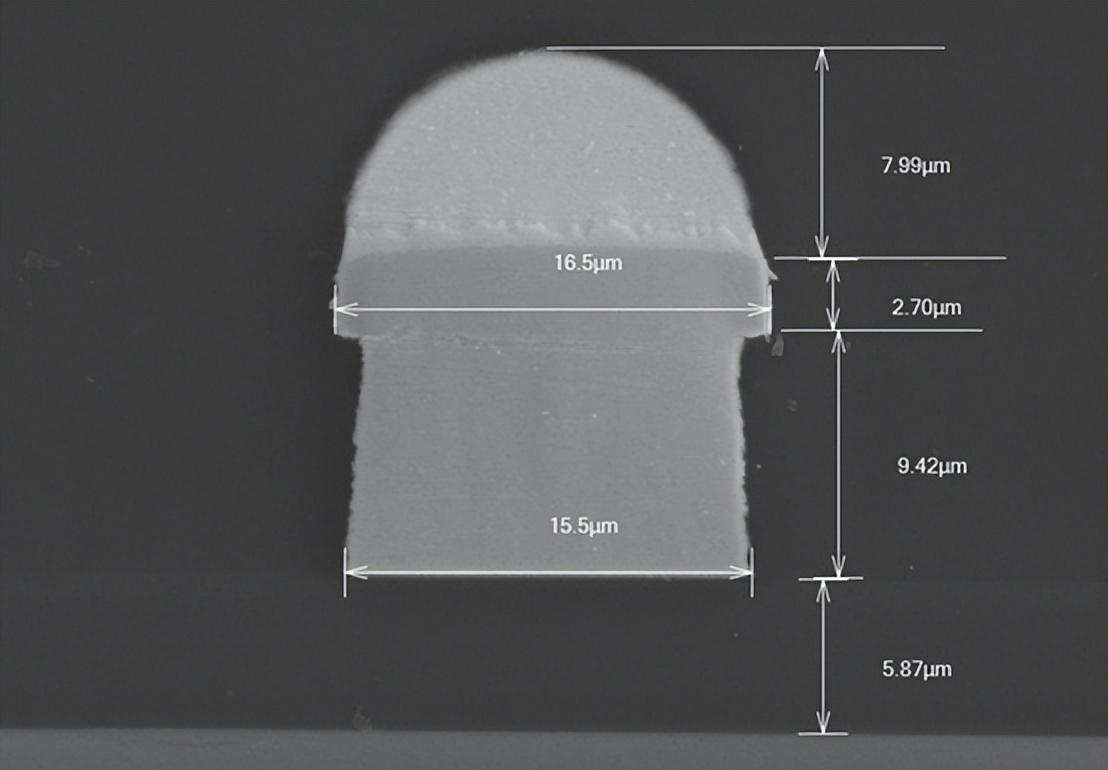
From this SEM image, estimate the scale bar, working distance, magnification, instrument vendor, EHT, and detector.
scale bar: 10000 nm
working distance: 14.4 mm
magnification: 3 K X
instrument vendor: Hitachi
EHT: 15 kV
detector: LA5(UL)